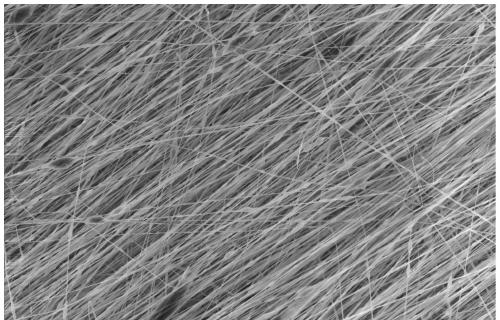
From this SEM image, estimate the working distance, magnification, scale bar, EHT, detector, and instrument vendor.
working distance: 9.9 mm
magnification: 1 K X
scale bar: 50000 nm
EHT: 10 kV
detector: SE(U)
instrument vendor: Hitachi